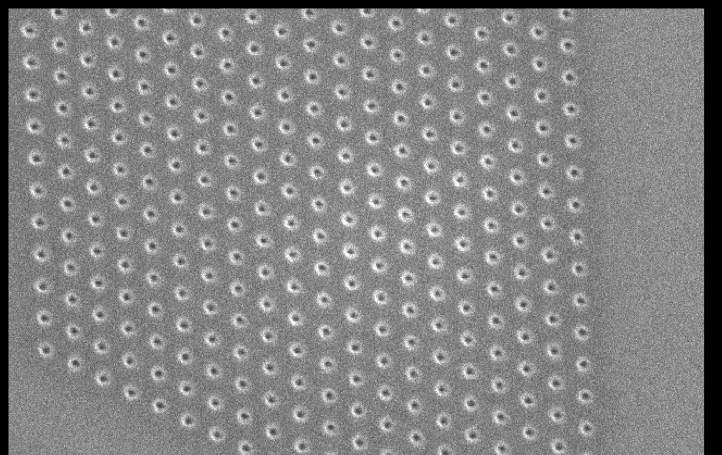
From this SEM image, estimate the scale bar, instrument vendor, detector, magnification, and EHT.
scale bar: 1000 nm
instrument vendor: JEOL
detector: SEI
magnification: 19 K X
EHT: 15 kV